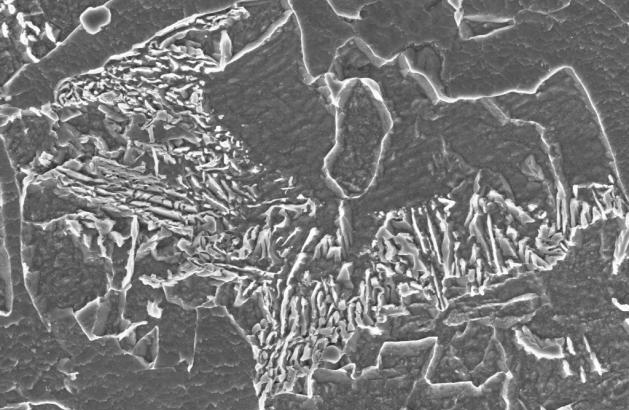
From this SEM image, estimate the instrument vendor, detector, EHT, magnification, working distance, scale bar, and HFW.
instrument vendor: Zeiss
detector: InLens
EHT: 5 kV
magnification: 30 K X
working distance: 3.1 mm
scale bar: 1000 nm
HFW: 9.932 µm